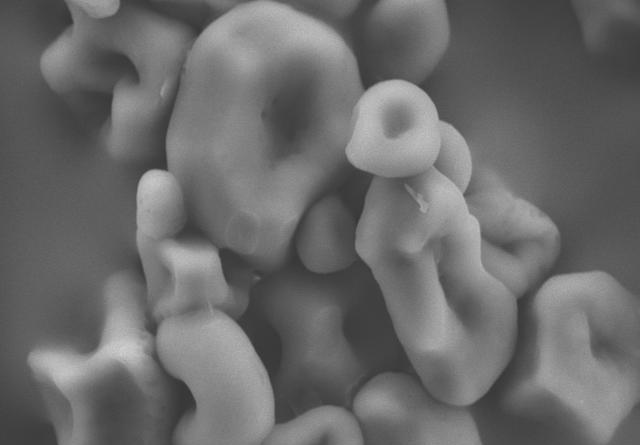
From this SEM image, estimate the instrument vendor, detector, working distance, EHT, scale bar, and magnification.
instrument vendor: Hitachi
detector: SE(M)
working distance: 16.6 mm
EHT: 15 kV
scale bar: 20000 nm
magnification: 2.5 K X